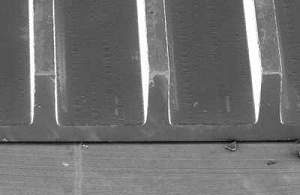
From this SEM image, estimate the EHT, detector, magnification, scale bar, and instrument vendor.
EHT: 10 kV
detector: SEI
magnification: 0.05 K X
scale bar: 500000 nm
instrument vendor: JEOL